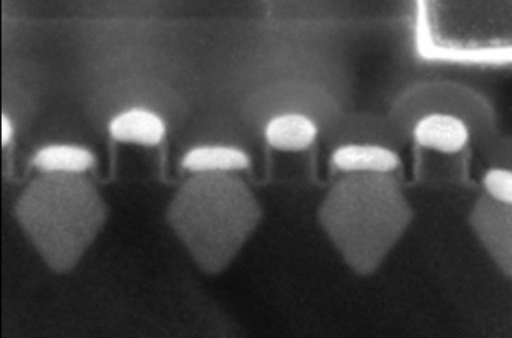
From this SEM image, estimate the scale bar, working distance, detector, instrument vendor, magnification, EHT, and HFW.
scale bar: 200 nm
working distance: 1.5 mm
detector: MD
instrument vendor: FEI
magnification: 300 K X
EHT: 2 kV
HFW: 0.423 µm